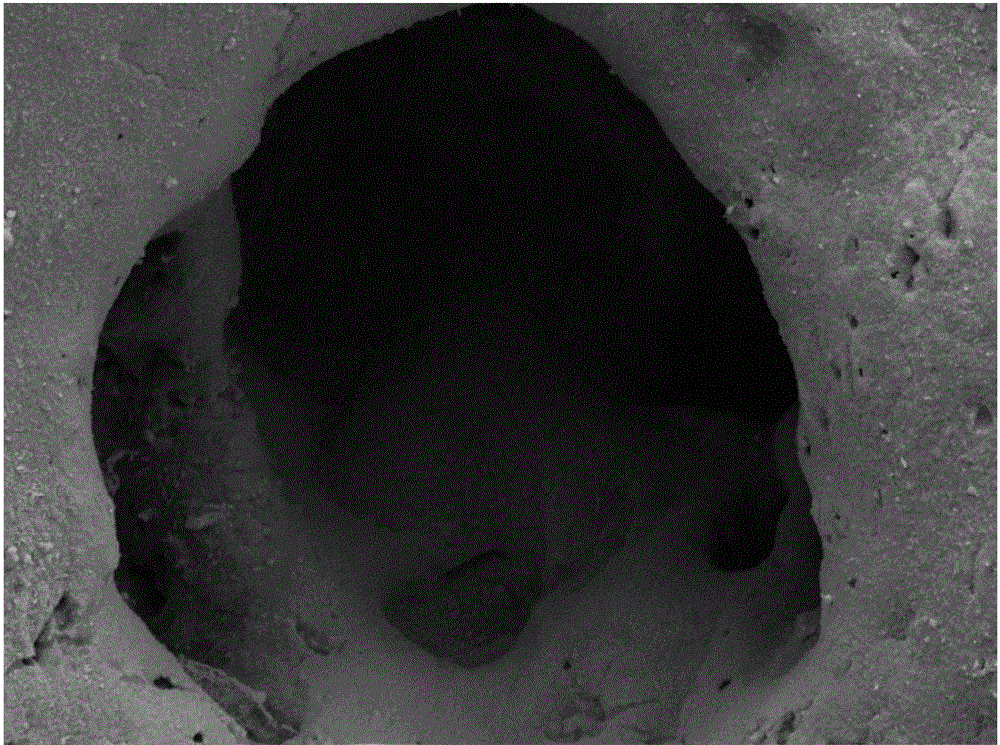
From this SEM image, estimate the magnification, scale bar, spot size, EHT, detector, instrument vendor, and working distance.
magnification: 1 K X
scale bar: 20000 nm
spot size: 4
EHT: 20 kV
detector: SE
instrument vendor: FEI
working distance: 22 mm